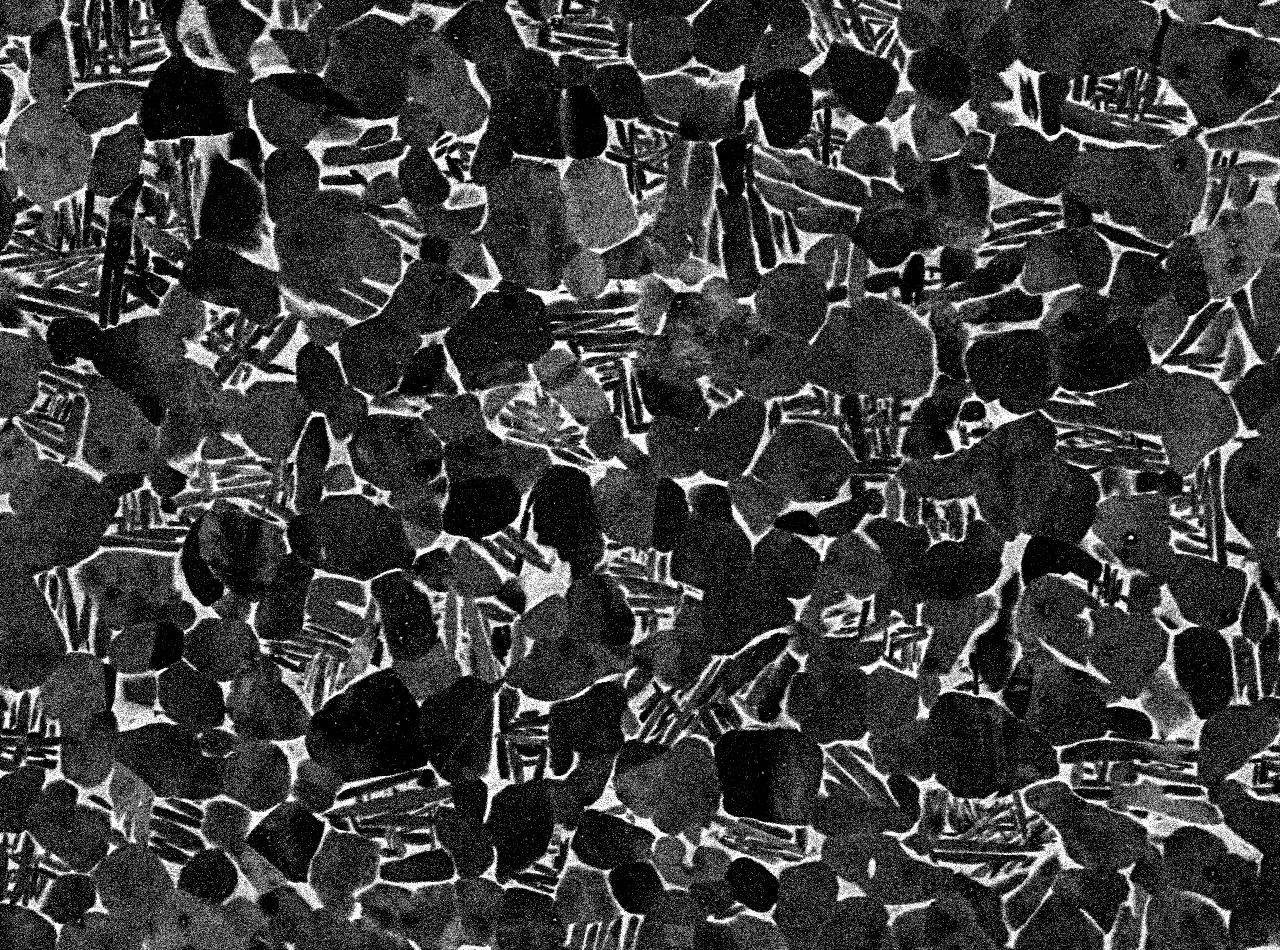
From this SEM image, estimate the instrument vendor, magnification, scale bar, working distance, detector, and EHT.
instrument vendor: JEOL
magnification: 1.1 K X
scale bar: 10000 nm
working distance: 10 mm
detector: COMPO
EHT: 15 kV